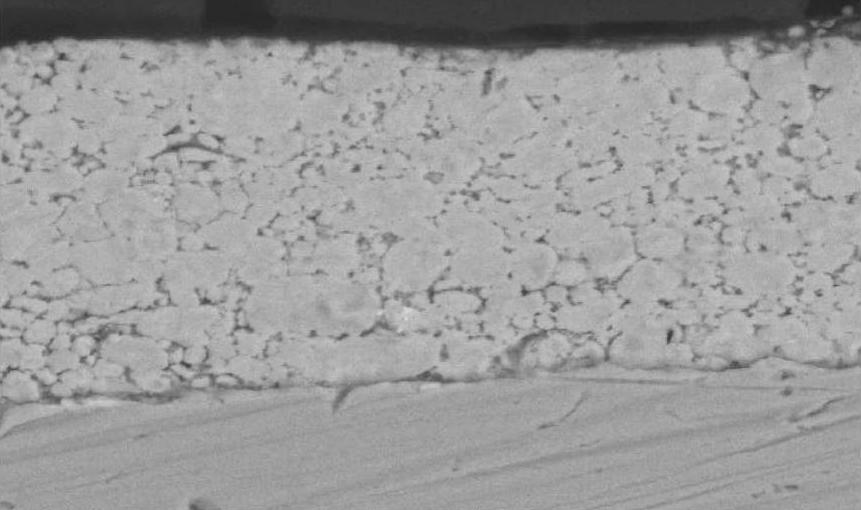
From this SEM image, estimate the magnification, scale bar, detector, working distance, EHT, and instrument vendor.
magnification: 2 K X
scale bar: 20000 nm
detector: BSE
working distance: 14.8 mm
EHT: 30 kV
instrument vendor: FEI